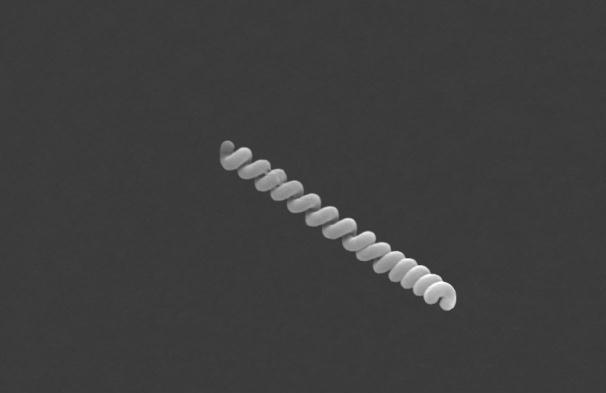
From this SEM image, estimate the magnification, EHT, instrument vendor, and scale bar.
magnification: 10 K X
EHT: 15 kV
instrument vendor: Zeiss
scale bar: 1000 nm